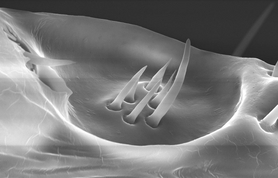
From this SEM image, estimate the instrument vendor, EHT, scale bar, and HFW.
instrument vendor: FEI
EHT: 20 kV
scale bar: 20000 nm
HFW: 69.3 µm